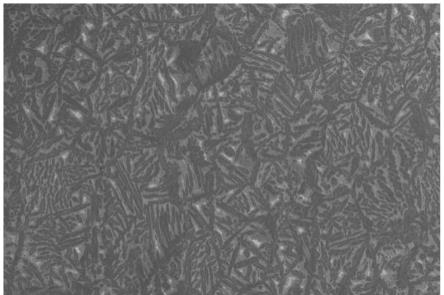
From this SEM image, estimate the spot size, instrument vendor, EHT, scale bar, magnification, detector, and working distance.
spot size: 60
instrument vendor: JEOL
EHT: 20 kV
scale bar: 100000 nm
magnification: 0.1 K X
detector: SEI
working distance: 12 mm